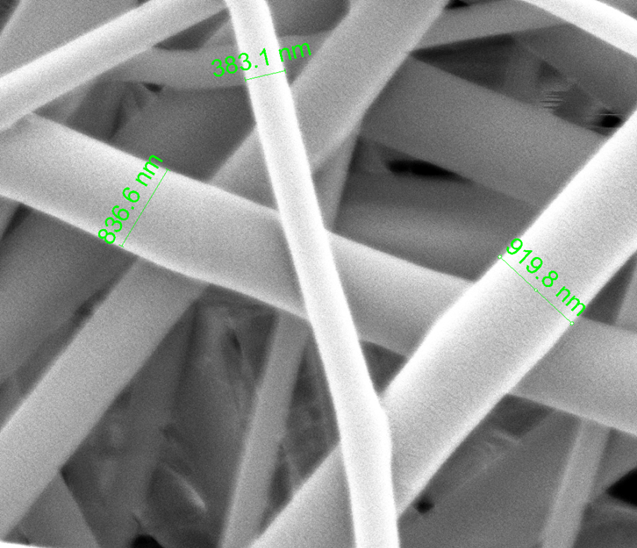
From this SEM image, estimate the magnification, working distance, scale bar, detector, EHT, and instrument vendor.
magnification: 50 K X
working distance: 10.3 mm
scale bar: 2000 nm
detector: ETD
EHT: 4 kV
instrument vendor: FEI